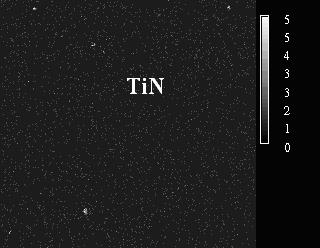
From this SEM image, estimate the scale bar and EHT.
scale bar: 50000 nm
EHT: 15 kV